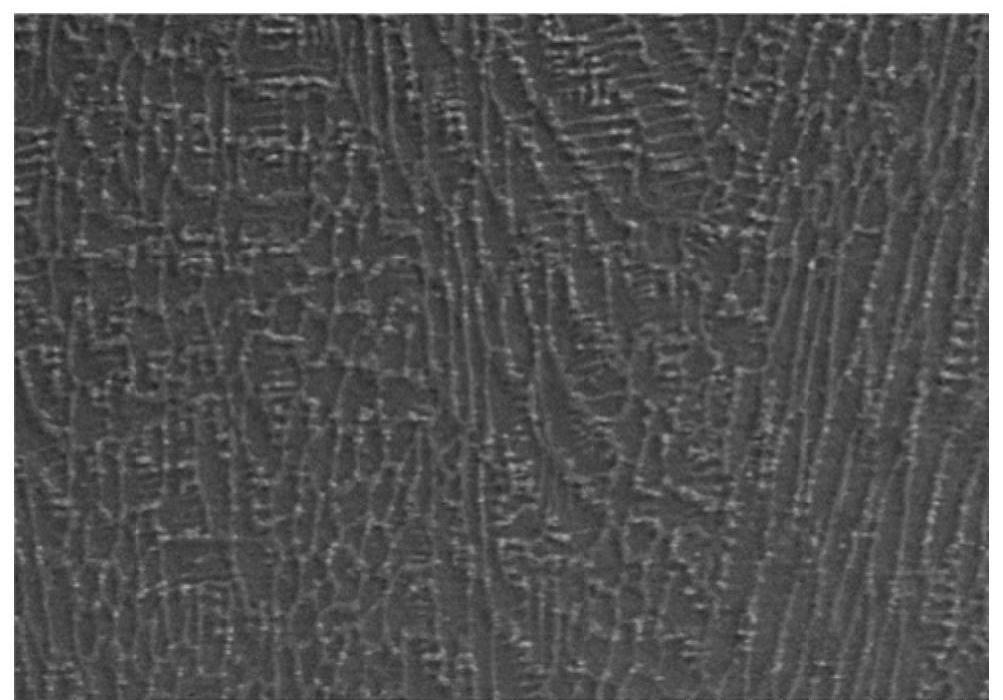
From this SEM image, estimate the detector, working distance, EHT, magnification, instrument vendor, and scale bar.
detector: SE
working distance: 7.2 mm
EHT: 15 kV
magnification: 2 K X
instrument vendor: Hitachi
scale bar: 20000 nm